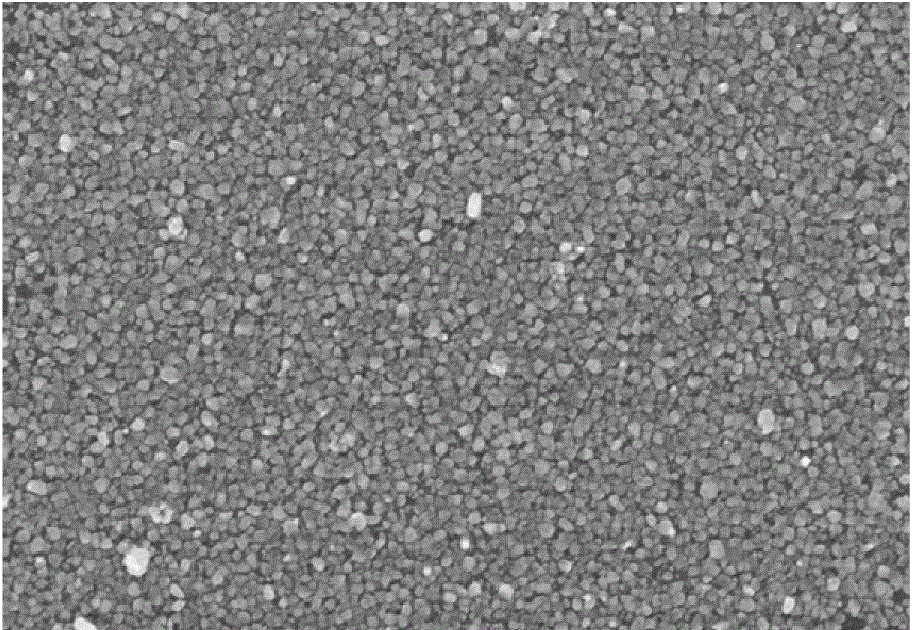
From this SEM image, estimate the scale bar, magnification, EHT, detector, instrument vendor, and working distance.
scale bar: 1000 nm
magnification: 30 K X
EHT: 10 kV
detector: SE(M)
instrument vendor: Hitachi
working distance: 10.2 mm